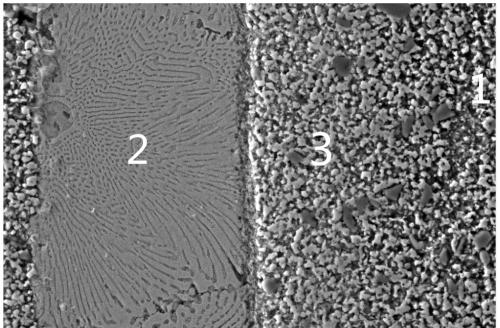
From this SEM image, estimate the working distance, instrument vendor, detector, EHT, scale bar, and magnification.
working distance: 8 mm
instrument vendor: Zeiss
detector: SE2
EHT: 20 kV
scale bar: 10000 nm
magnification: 0.815 K X